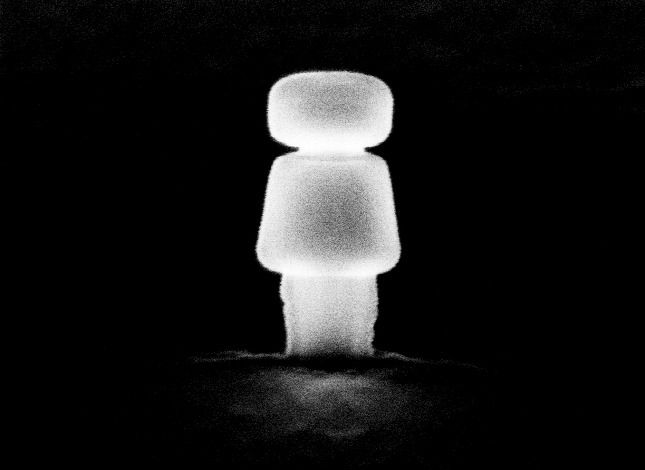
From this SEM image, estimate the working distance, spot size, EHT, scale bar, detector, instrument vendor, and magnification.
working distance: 18.3 mm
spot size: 3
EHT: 10 kV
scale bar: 500 nm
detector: SE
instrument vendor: FEI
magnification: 42.123 K X